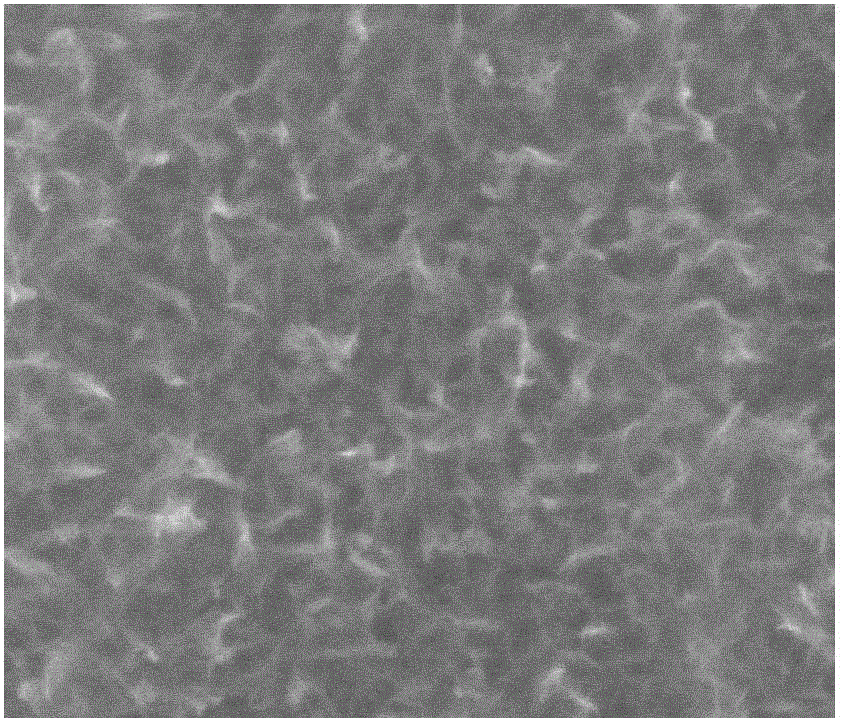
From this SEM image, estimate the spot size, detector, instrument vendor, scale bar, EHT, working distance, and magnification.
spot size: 3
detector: LFD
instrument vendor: FEI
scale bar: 500 nm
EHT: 20 kV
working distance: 10 mm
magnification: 120 K X